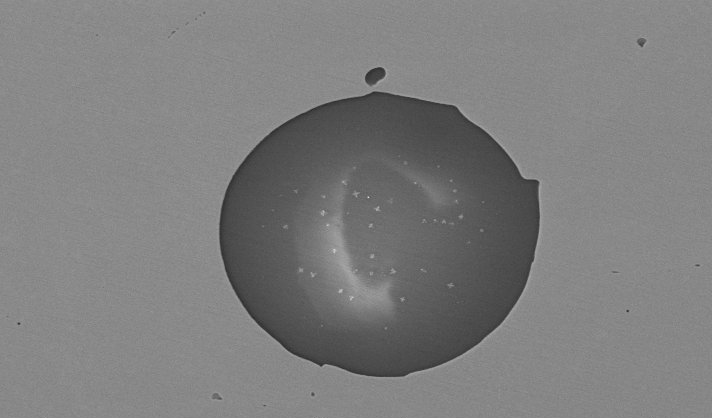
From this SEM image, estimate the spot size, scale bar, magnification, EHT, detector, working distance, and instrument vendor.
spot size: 4.4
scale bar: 20000 nm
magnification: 1.5 K X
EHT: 20 kV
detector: BSE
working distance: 9 mm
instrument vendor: FEI